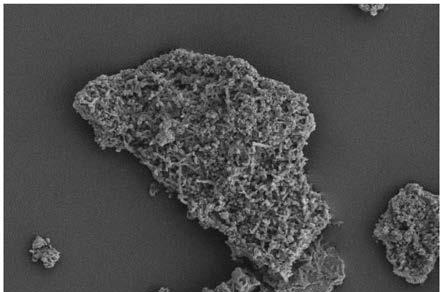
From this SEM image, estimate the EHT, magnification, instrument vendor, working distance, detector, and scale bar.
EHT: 10 kV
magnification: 1 K X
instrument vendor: Zeiss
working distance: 8.9 mm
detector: SE2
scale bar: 20000 nm